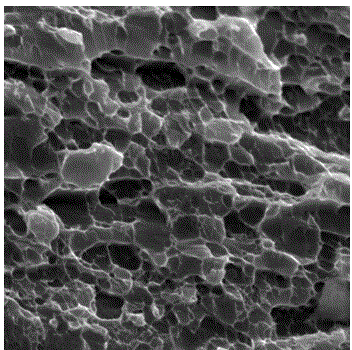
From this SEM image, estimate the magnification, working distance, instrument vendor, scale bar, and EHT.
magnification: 7 K X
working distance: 11.54 mm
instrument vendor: Tescan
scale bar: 5000 nm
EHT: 20 kV